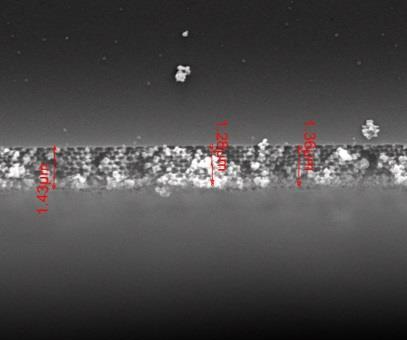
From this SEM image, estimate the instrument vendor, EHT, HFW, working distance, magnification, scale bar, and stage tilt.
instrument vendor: FEI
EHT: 25 kV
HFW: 13.5 µm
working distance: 9.9 mm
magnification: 20 K X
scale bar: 5000 nm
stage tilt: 0°